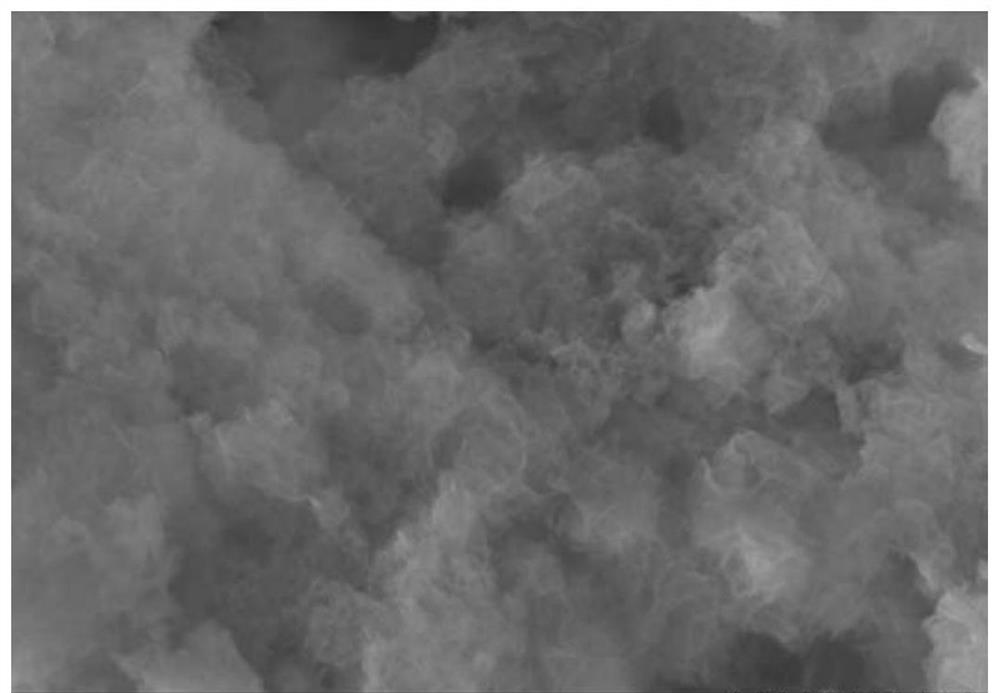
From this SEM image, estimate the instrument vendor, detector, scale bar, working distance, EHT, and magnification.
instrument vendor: Hitachi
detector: SE(M)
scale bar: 500 nm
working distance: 4.1 mm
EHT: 5 kV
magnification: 60 K X